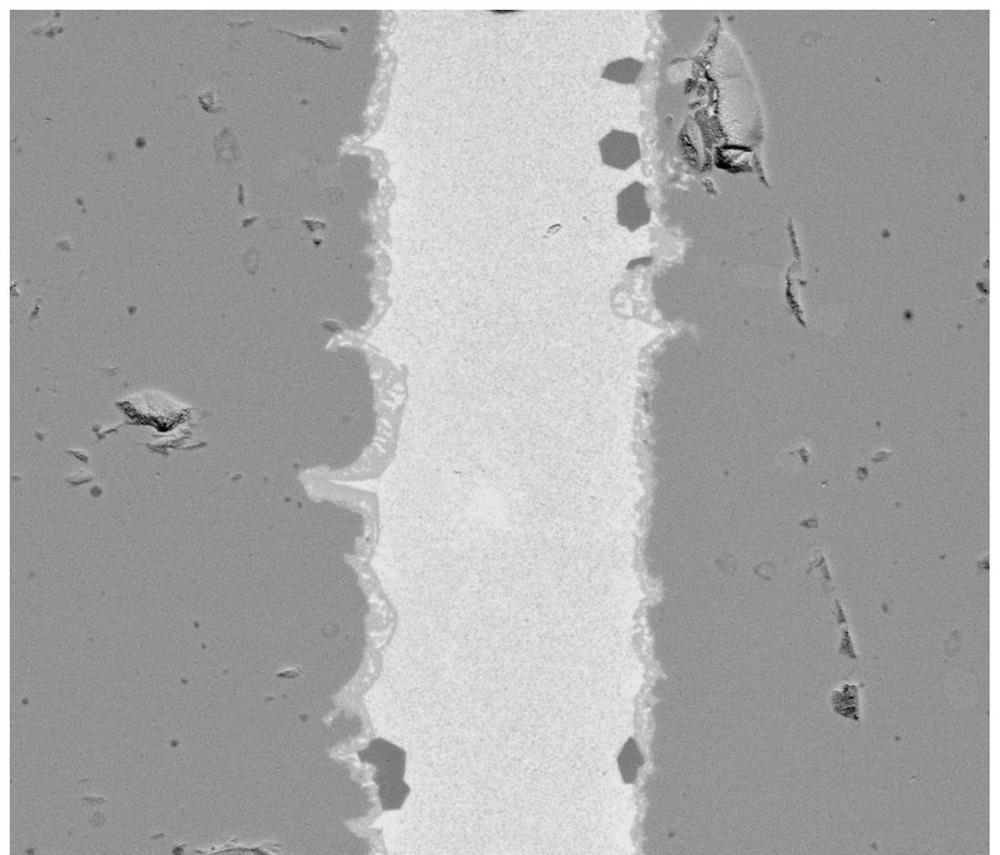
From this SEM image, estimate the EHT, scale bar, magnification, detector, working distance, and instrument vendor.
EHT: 20 kV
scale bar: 50000 nm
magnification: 2.5 K X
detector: Z Cont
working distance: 14.4 mm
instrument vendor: FEI